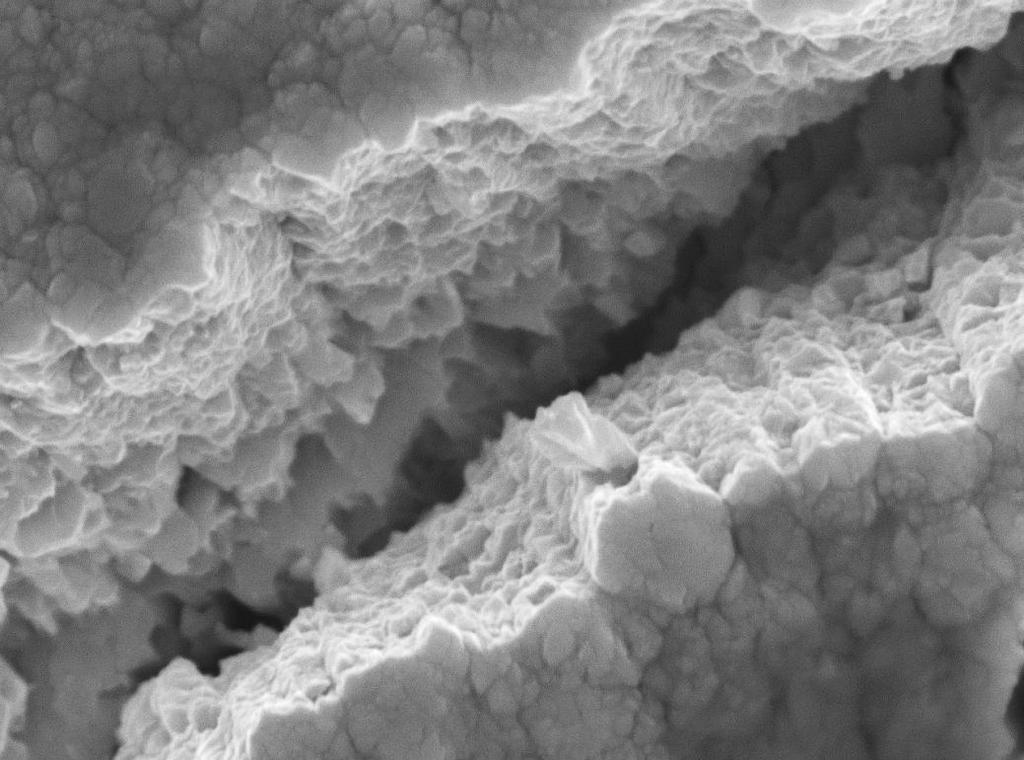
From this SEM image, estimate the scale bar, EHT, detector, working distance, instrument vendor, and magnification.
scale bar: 100 nm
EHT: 15 kV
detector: SEI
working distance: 10.7 mm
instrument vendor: JEOL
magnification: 30 K X